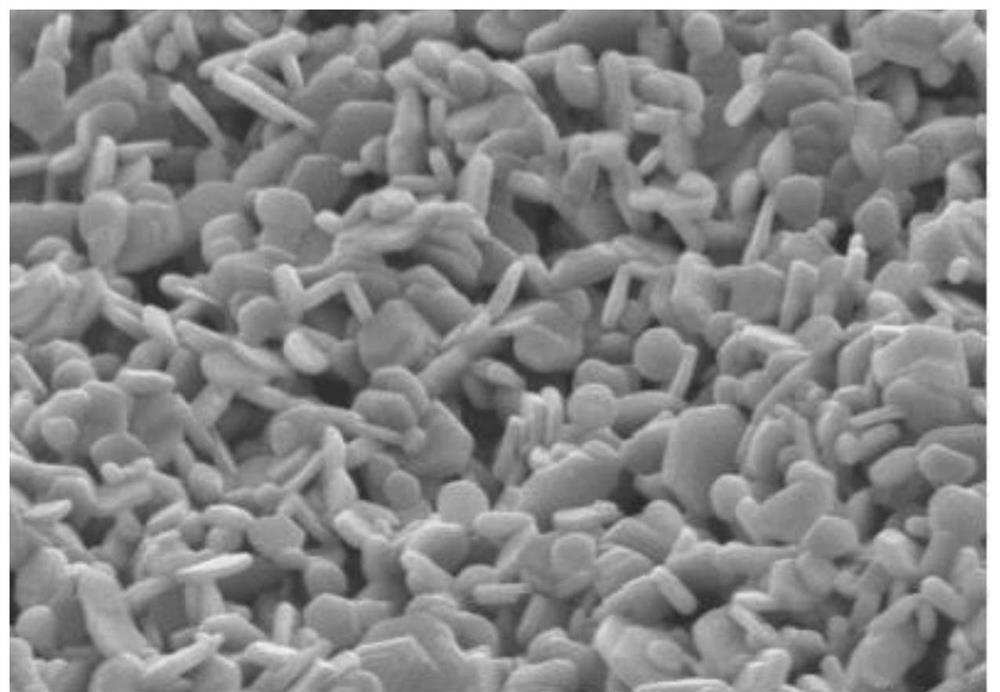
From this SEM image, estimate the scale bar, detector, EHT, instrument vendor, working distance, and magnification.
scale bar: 10000 nm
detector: SE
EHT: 15 kV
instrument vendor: Hitachi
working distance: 15.5 mm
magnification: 5 K X